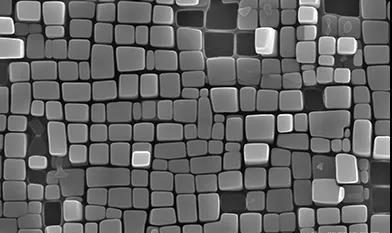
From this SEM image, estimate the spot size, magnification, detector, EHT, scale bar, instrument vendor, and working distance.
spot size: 3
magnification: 60 K X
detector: ETD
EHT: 15 kV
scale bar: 1000 nm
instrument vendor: FEI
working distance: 12.3 mm